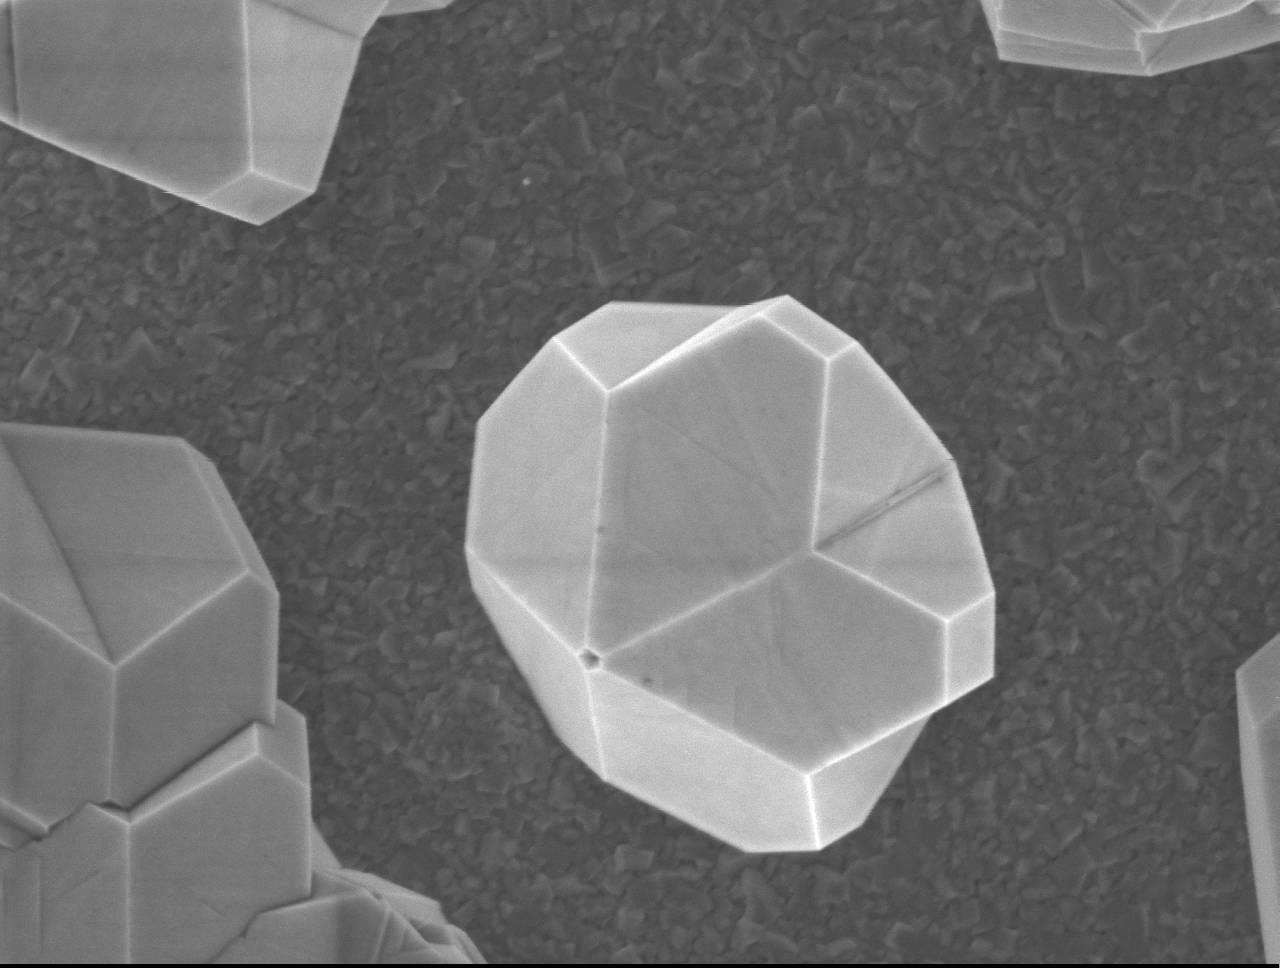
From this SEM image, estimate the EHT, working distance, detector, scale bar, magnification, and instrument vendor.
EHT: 3 kV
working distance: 7.5 mm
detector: SEI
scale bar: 100 nm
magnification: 30 K X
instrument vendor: JEOL